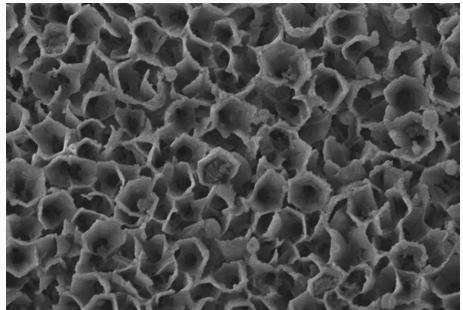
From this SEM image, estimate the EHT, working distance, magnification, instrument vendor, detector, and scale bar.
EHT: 5 kV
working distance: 8.4 mm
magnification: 5 K X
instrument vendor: Hitachi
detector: SE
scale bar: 10000 nm